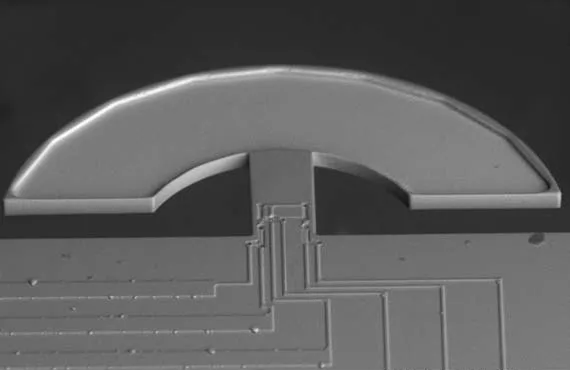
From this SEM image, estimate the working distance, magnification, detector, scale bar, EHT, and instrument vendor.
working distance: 12.3 mm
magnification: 0.32 K X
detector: BSE3D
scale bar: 100000 nm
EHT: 15 kV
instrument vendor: Hitachi